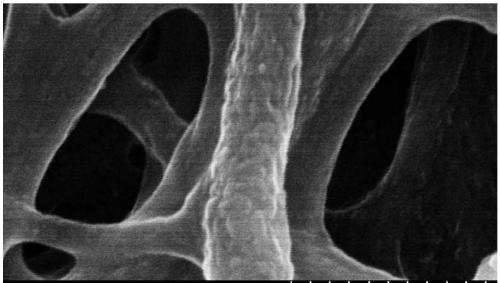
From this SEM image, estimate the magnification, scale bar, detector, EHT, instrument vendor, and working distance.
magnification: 100 K X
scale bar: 500 nm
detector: SE(M,LA0)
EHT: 10 kV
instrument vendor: Hitachi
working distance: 9.6 mm